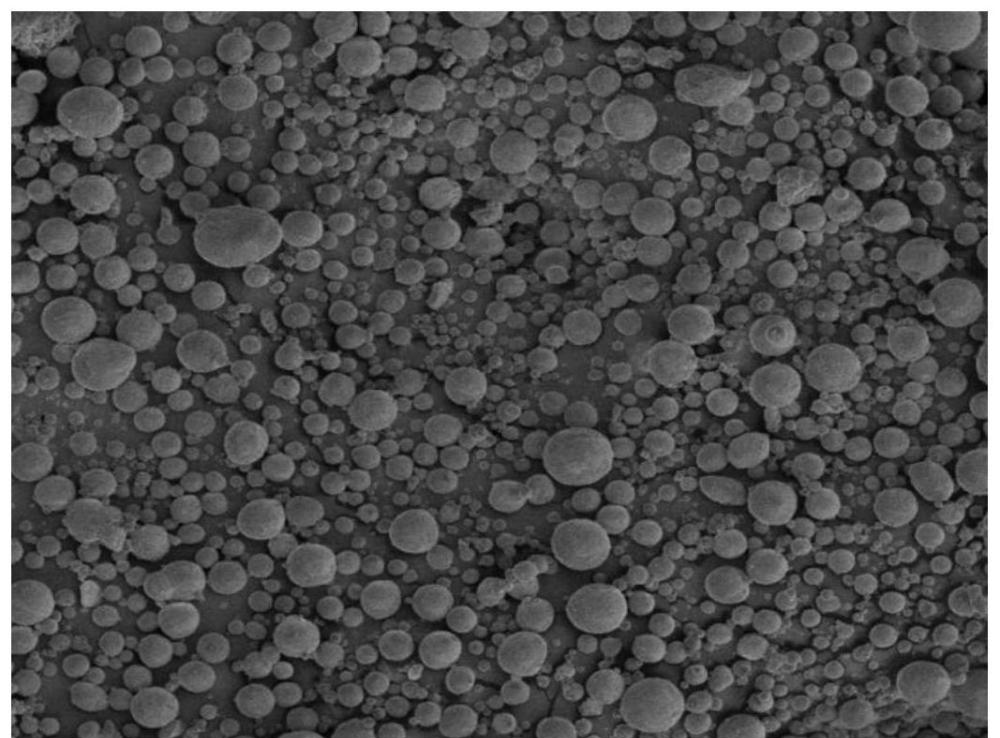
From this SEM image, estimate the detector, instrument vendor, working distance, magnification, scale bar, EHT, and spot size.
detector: ETD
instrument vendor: FEI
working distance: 4.7 mm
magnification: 0.5 K X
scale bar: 100000 nm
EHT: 5 kV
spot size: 3.5